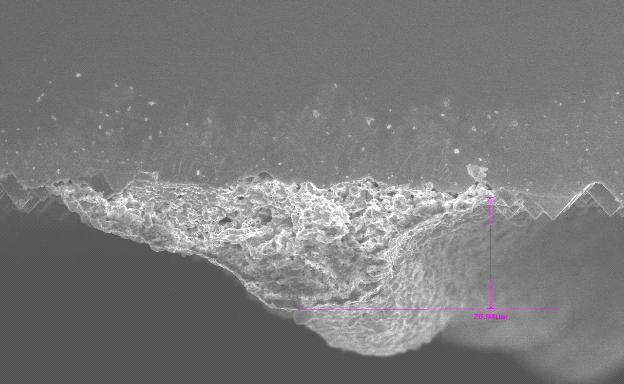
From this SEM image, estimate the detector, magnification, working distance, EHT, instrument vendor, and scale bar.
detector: SEI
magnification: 0.95 K X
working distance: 8 mm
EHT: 10 kV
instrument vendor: JEOL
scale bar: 10000 nm